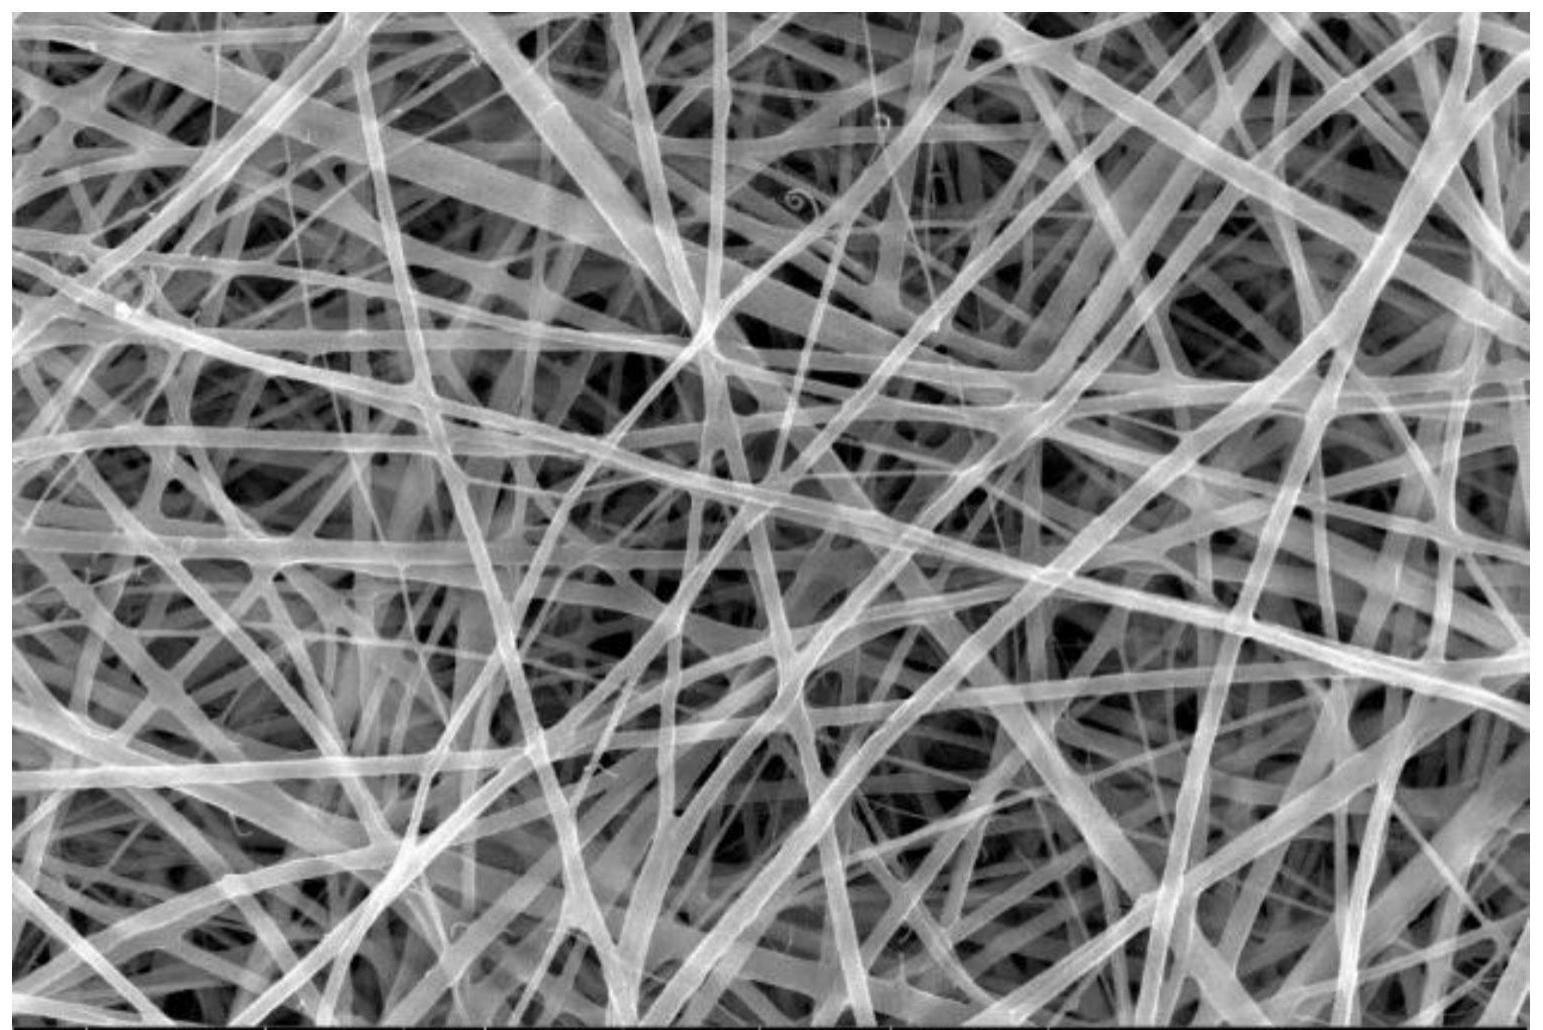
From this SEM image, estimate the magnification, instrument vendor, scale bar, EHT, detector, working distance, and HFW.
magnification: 48 K X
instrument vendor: FEI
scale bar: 2000 nm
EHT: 20 kV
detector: ETD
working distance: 13.9 mm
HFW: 8.63 µm